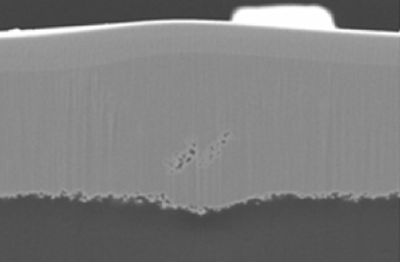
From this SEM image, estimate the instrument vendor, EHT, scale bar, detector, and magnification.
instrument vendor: JEOL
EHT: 15 kV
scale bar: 10000 nm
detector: SEI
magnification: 2.5 K X